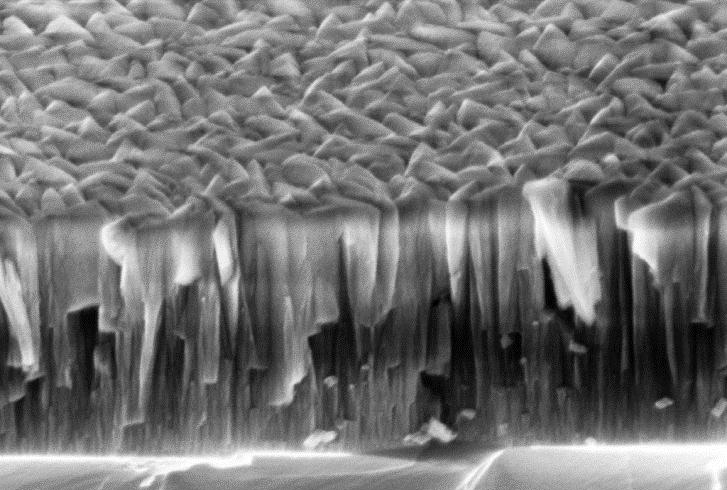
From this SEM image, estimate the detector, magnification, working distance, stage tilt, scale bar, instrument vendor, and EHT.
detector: InLens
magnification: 140 K X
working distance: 4.5 mm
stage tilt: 30°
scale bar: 200 nm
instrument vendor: Zeiss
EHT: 5 kV